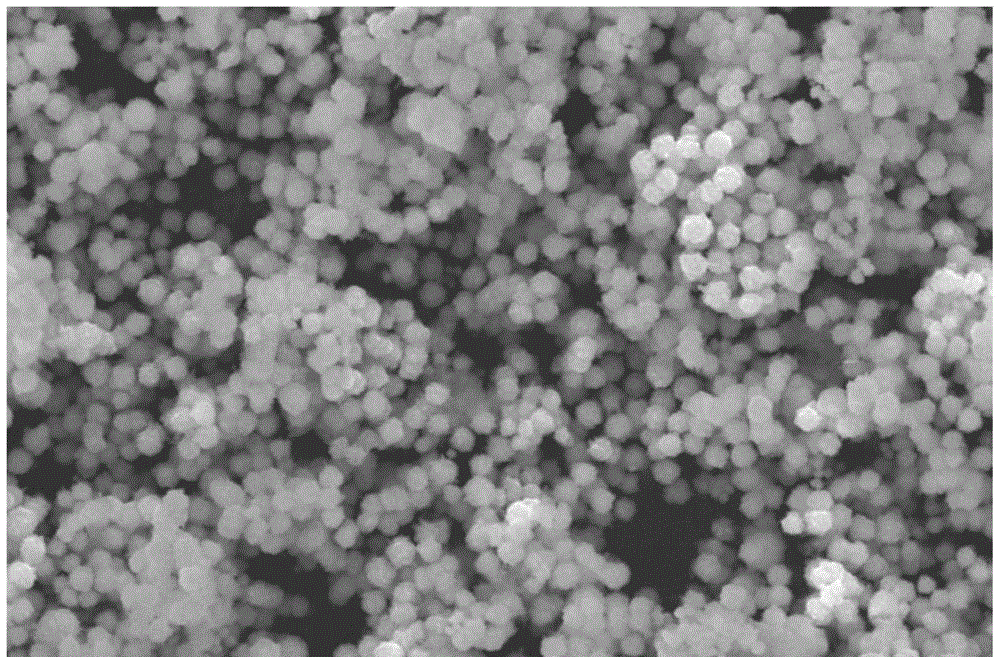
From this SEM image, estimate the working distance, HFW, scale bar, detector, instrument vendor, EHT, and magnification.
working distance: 10 mm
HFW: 20.7 µm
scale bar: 5000 nm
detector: ETD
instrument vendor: FEI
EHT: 20 kV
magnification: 20 K X